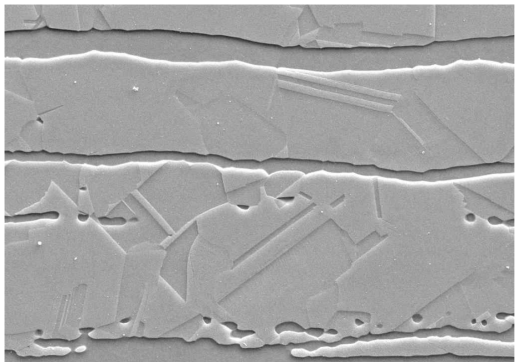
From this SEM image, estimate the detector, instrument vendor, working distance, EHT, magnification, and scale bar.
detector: SE2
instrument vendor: Zeiss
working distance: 10.9 mm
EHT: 15 kV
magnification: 3 K X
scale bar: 1000 nm